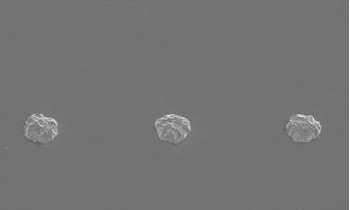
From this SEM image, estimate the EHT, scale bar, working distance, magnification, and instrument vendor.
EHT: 15 kV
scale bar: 200000 nm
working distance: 21.8 mm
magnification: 0.2 K X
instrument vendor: Hitachi